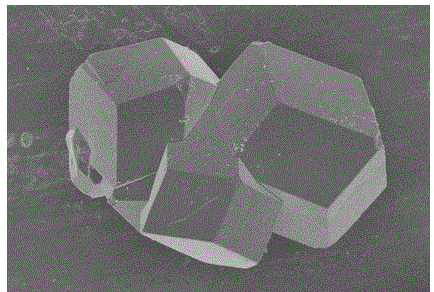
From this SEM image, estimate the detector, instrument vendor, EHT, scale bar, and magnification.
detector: SE(U)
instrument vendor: Hitachi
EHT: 4 kV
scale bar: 50000 nm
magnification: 1 K X